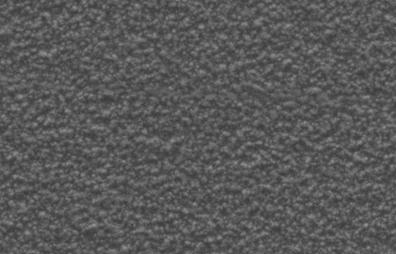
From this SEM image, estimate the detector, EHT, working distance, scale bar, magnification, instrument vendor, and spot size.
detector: SE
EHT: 5 kV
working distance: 6.9 mm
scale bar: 1000 nm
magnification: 50 K X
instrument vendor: FEI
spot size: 3